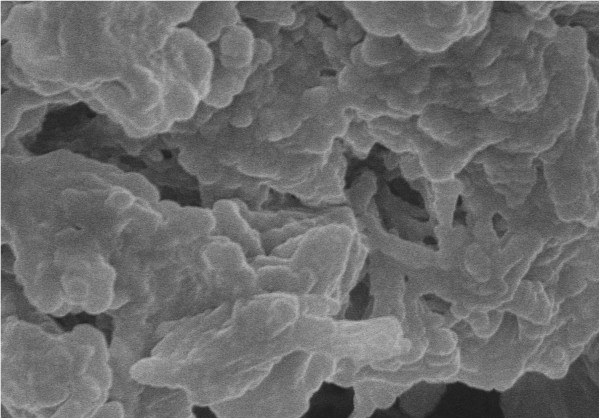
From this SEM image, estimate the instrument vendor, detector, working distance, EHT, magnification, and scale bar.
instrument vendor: Hitachi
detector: SE(U)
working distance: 12.4 mm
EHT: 10 kV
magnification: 8.01 K X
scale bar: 5000 nm